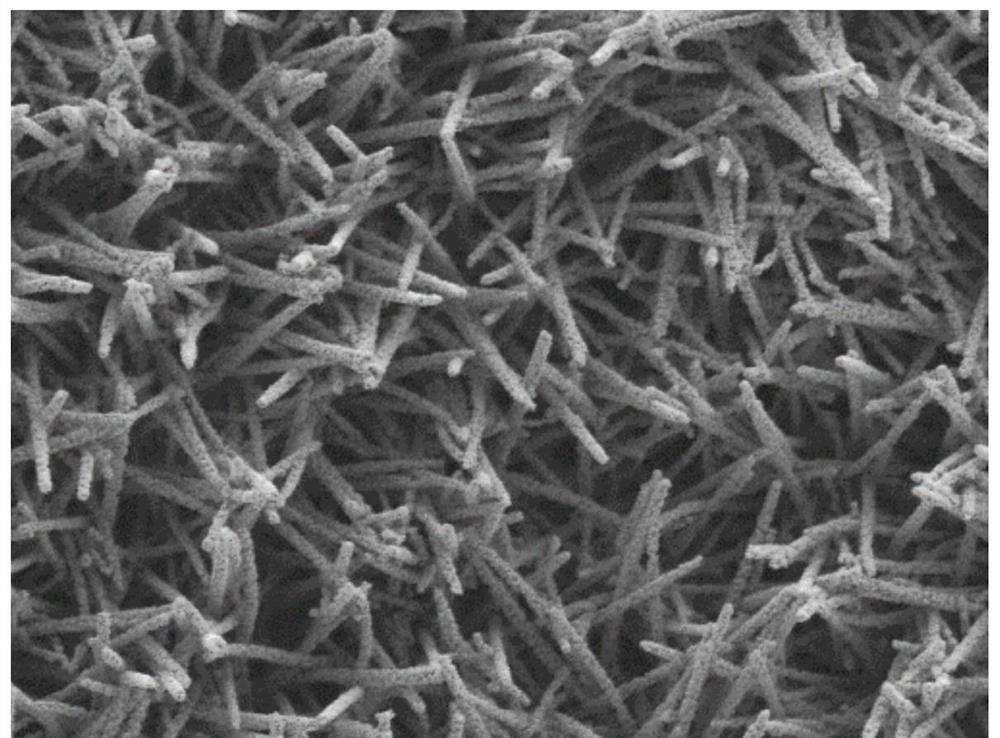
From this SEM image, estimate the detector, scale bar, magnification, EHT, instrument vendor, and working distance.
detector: LED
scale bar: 1000 nm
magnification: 10 K X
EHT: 3 kV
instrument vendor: JEOL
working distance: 8 mm